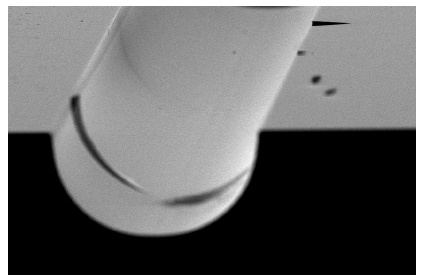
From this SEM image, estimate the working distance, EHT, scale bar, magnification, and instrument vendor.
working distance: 11.2 mm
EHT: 20 kV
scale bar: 100000 nm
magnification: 0.5 K X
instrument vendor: FEI